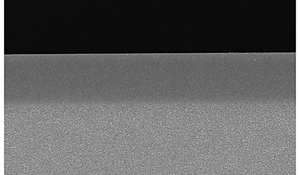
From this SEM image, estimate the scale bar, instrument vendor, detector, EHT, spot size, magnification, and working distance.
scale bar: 2000 nm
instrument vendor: FEI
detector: TLD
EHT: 5 kV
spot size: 3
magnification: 15 K X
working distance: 4.7 mm